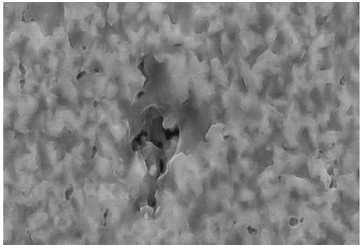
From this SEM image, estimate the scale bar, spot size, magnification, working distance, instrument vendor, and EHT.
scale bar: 1000 nm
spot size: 8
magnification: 30 K X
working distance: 5 mm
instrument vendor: other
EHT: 20 kV